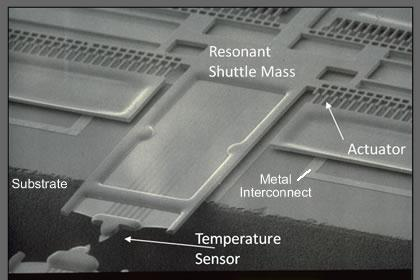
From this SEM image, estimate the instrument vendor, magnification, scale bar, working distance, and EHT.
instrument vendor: JEOL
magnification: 0.25 K X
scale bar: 100000 nm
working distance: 28 mm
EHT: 10 kV